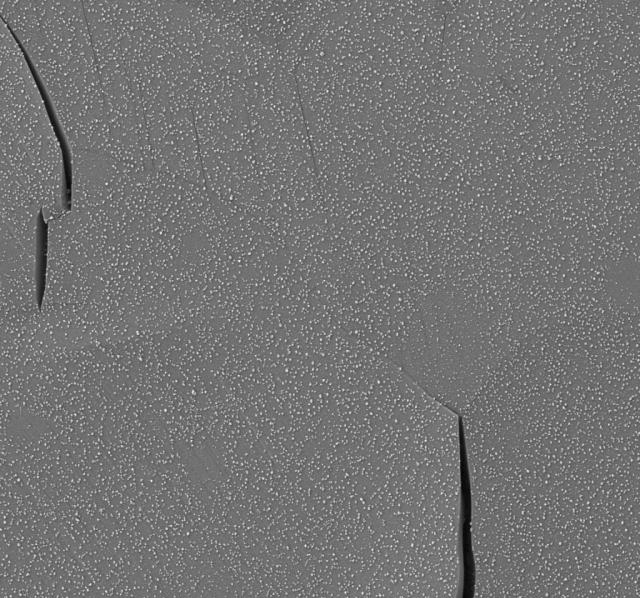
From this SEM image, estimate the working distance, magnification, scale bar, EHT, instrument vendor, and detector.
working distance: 9.7 mm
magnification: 2.17 K X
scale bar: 4000 nm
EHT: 5 kV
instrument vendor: Zeiss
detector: SE2